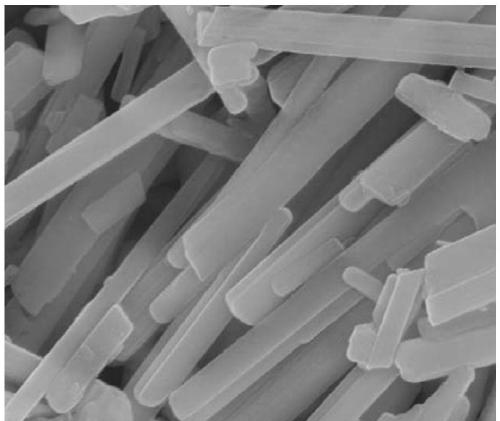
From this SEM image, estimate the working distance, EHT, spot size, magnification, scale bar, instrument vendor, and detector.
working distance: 5.9 mm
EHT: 10 kV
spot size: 3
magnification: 100 K X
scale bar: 500 nm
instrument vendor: FEI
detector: TLD